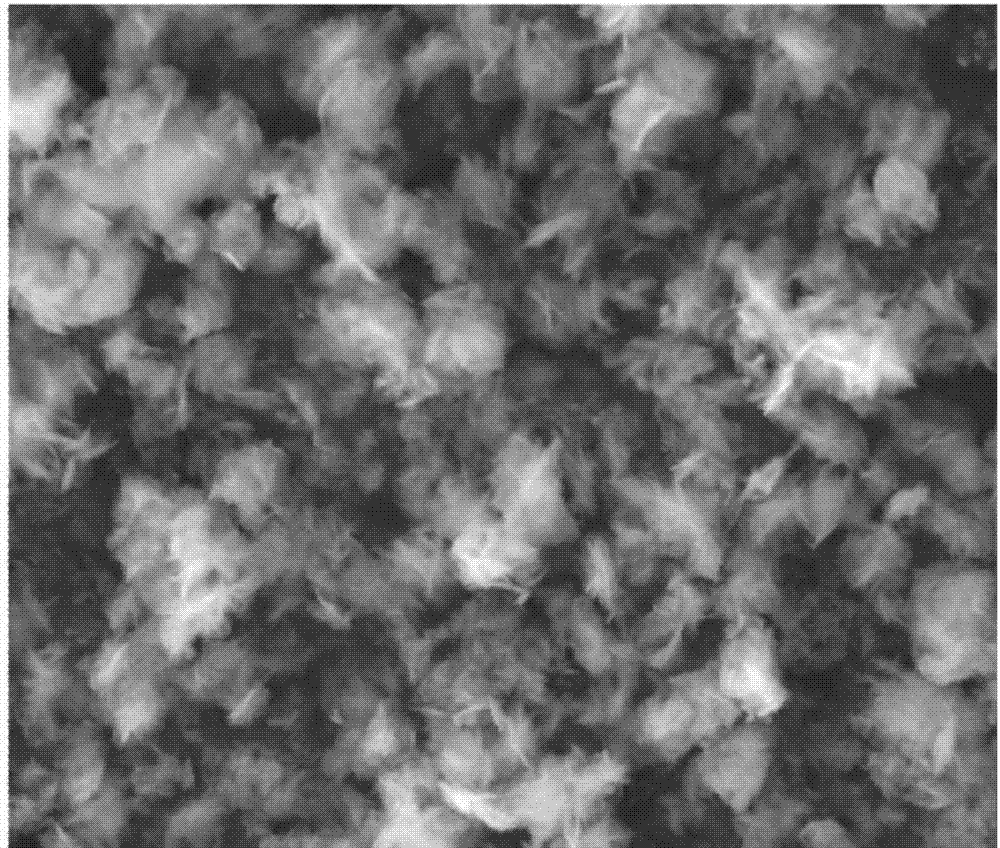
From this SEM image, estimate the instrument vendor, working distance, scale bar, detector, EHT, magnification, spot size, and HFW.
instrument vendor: FEI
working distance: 5 mm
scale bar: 5000 nm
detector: ETD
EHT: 15 kV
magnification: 15.037 K X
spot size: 5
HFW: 19.8 µm